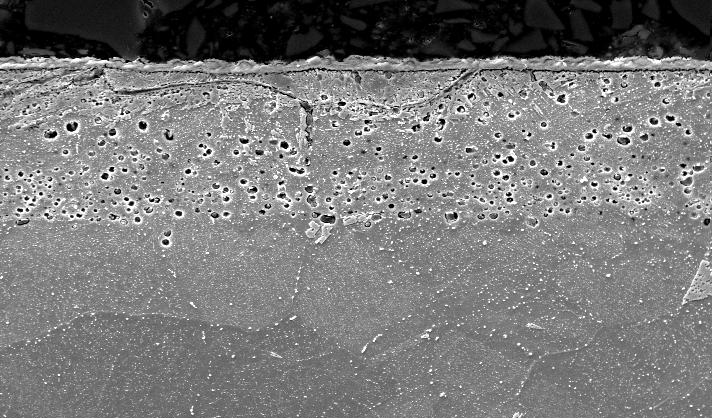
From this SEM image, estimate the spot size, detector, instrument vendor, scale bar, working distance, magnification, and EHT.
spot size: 5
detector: SE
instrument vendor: FEI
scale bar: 20000 nm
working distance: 10.4 mm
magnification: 1.5 K X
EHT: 20 kV